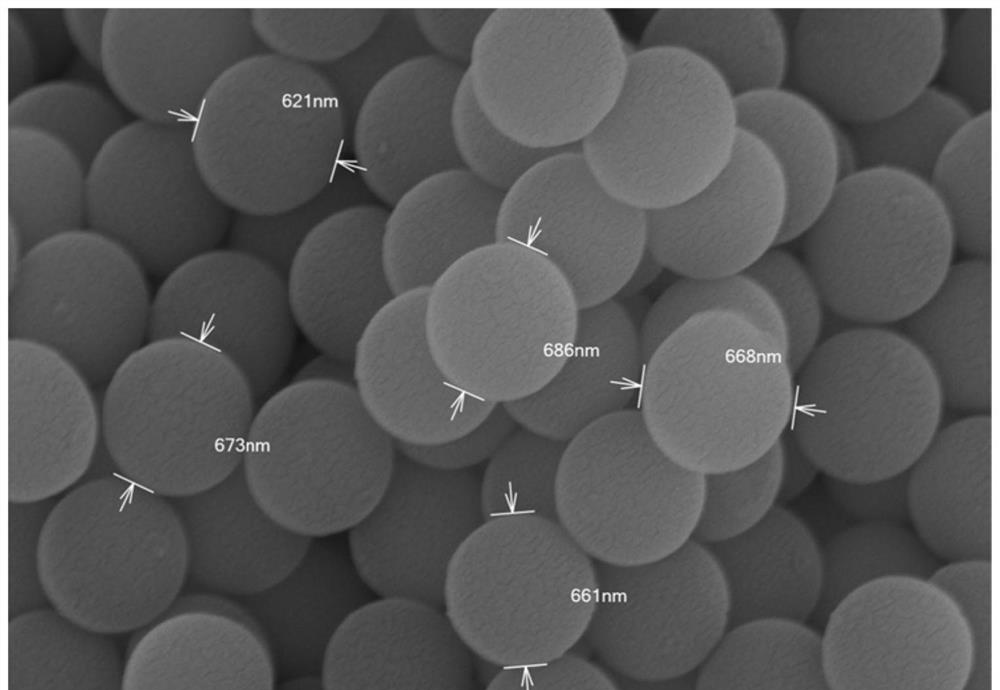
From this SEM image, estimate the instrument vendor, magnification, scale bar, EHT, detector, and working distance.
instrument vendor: Hitachi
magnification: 30 K X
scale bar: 1000 nm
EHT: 15 kV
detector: SE(L)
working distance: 6.1 mm